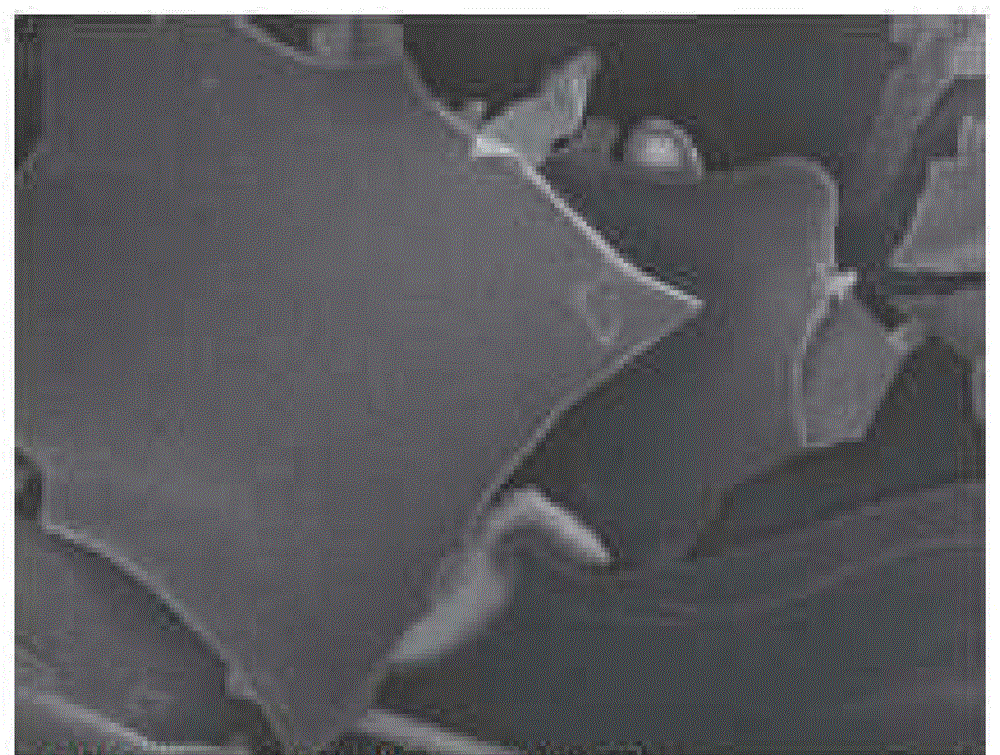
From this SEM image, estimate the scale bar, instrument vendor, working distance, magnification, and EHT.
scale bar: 10000 nm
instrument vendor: JEOL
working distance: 7.2 mm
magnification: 1 K X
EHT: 5 kV